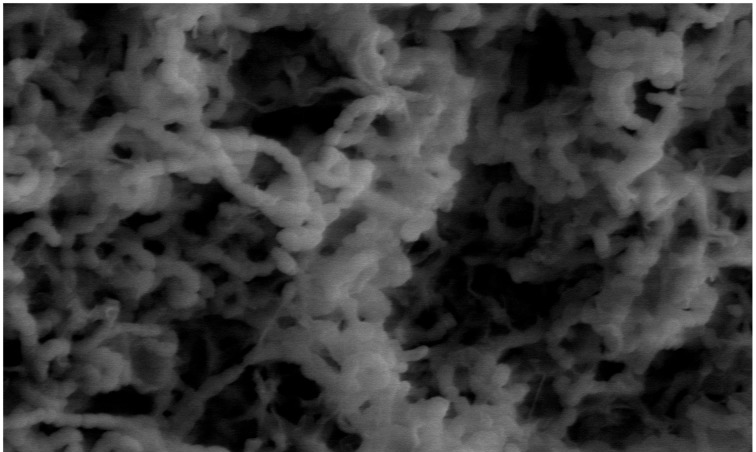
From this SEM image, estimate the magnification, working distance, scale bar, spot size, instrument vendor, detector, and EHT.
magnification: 8 K X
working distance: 11 mm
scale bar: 10000 nm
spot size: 3.5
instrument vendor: FEI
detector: LVD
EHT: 10 kV